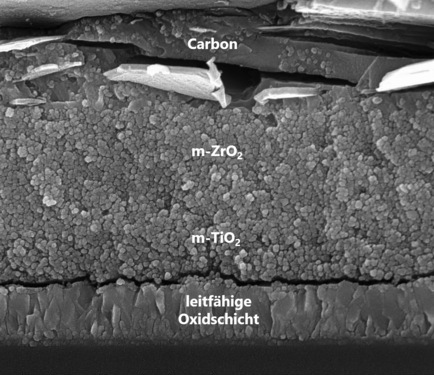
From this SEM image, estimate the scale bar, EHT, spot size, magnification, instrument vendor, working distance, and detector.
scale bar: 2000 nm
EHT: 15 kV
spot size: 3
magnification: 66.237 K X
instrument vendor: FEI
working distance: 5.2 mm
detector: TLD